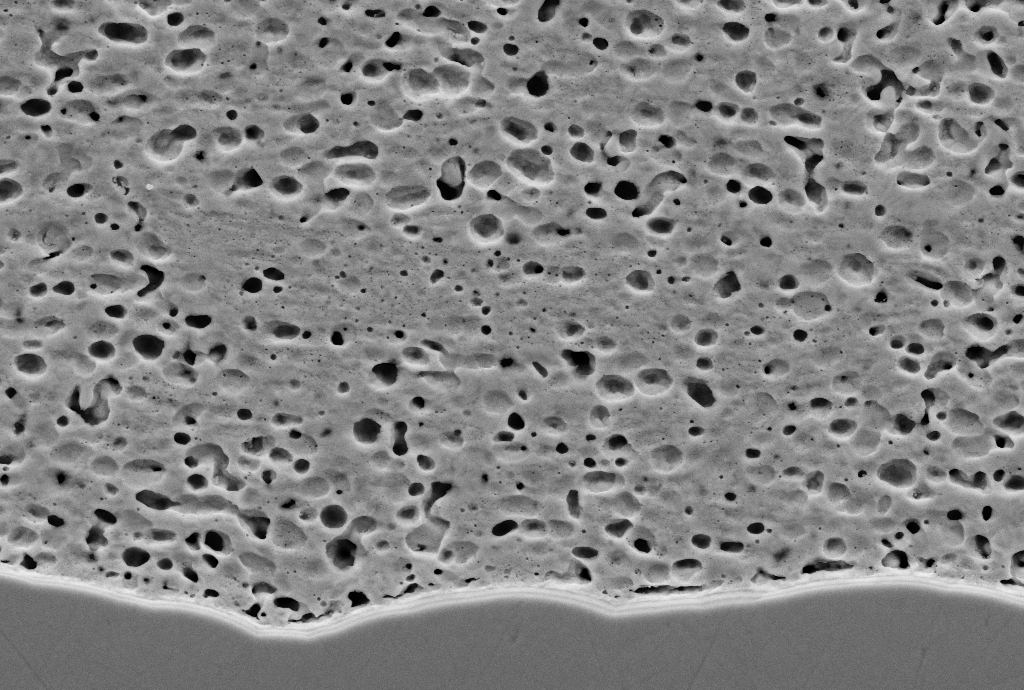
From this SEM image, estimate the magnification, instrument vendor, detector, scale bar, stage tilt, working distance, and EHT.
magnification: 5 K X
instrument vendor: Zeiss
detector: SE2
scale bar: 2000 nm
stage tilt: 0°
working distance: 7.9 mm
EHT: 10 kV